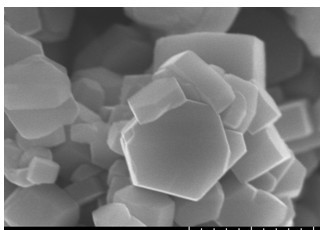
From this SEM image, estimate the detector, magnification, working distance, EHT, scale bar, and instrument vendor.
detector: SE(U)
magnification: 50 K X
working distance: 9.8 mm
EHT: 10 kV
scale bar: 1000 nm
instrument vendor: Hitachi